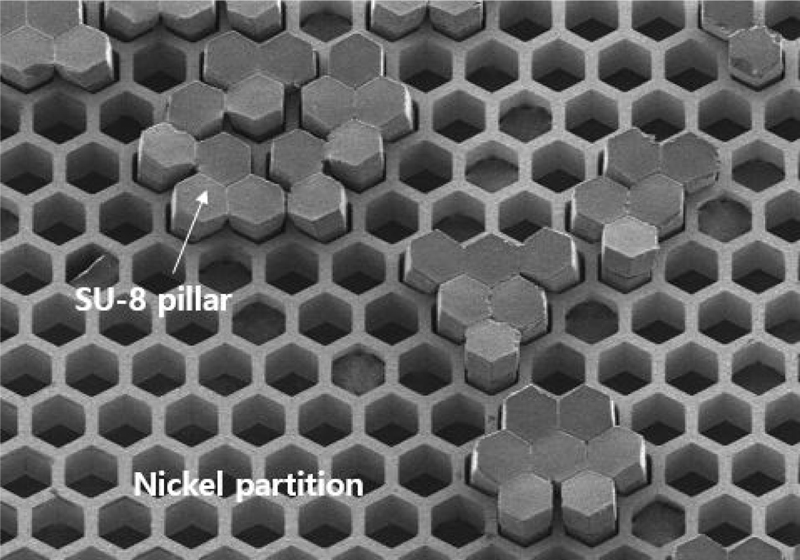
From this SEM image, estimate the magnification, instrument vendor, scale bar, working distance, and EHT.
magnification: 0.1 K X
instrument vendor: Hitachi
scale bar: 500000 nm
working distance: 8.8 mm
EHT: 5 kV